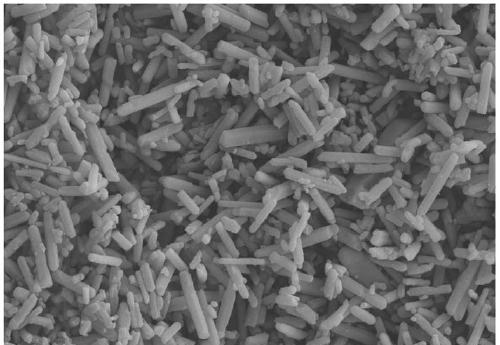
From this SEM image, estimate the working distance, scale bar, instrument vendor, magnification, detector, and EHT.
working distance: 7.2 mm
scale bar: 2000 nm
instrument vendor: Zeiss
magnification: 3 K X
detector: SE2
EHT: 15 kV